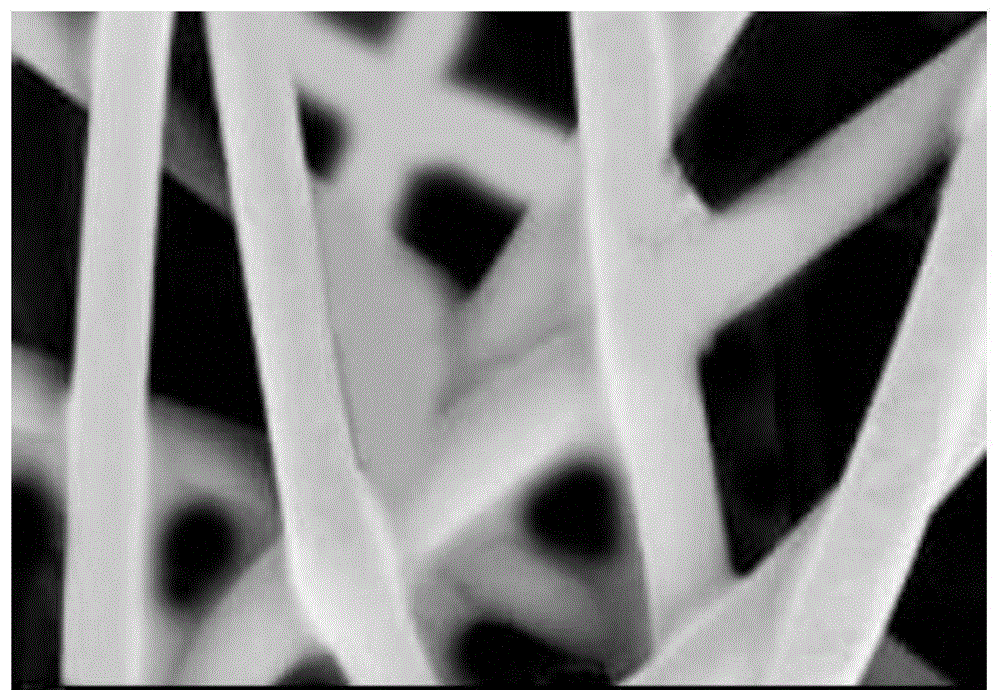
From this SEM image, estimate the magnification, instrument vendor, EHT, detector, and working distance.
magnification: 0.2 K X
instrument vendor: Zeiss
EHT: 3 kV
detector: SE2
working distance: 11 mm